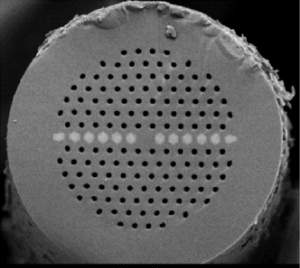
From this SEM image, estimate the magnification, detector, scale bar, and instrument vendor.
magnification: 0.55 K X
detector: BES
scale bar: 20000 nm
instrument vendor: JEOL